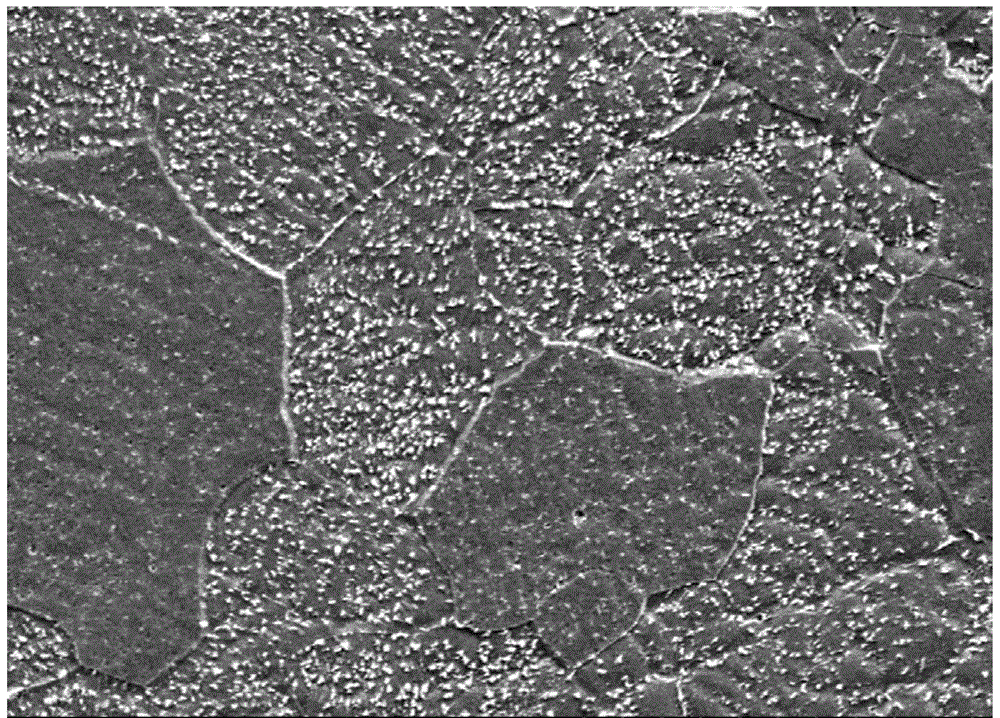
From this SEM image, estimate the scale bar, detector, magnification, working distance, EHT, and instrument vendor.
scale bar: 100000 nm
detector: SE(M)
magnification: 0.5 K X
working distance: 7.6 mm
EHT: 15 kV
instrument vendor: Hitachi